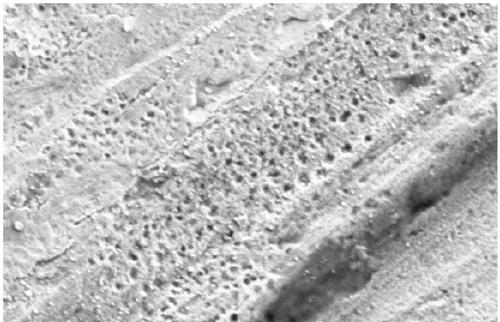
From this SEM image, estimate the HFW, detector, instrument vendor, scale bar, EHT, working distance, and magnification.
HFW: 6.35 µm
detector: ETD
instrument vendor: Thermo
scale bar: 2000 nm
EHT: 2 kV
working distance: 10 mm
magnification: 20 K X